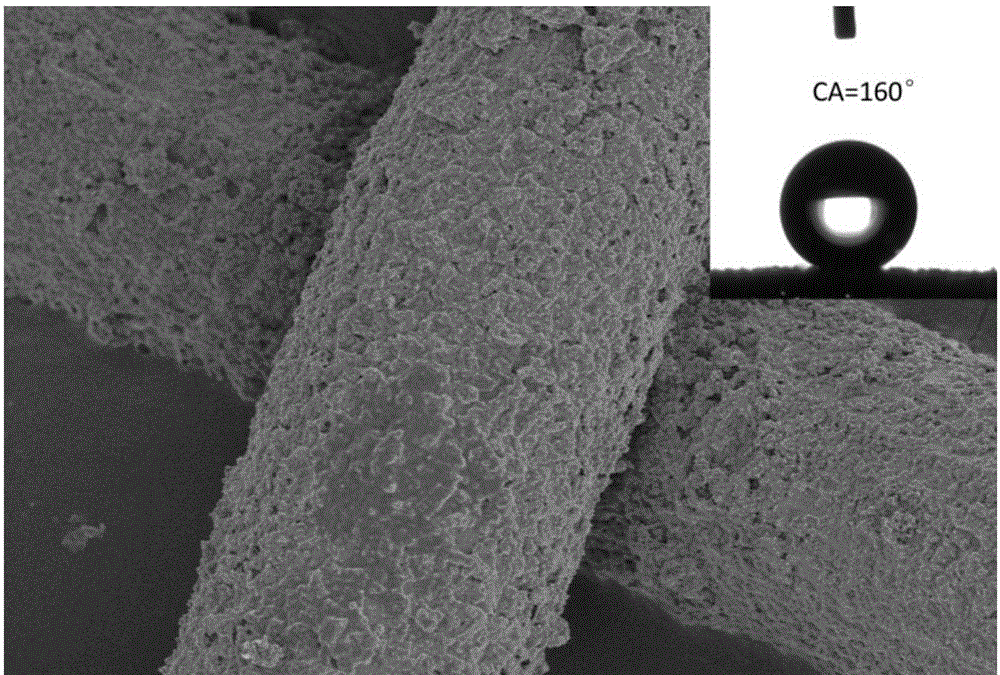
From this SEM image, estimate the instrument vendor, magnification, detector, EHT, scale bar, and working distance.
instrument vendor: Hitachi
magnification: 0.9 K X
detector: SE(M)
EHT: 5 kV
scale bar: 50000 nm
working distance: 11.2 mm